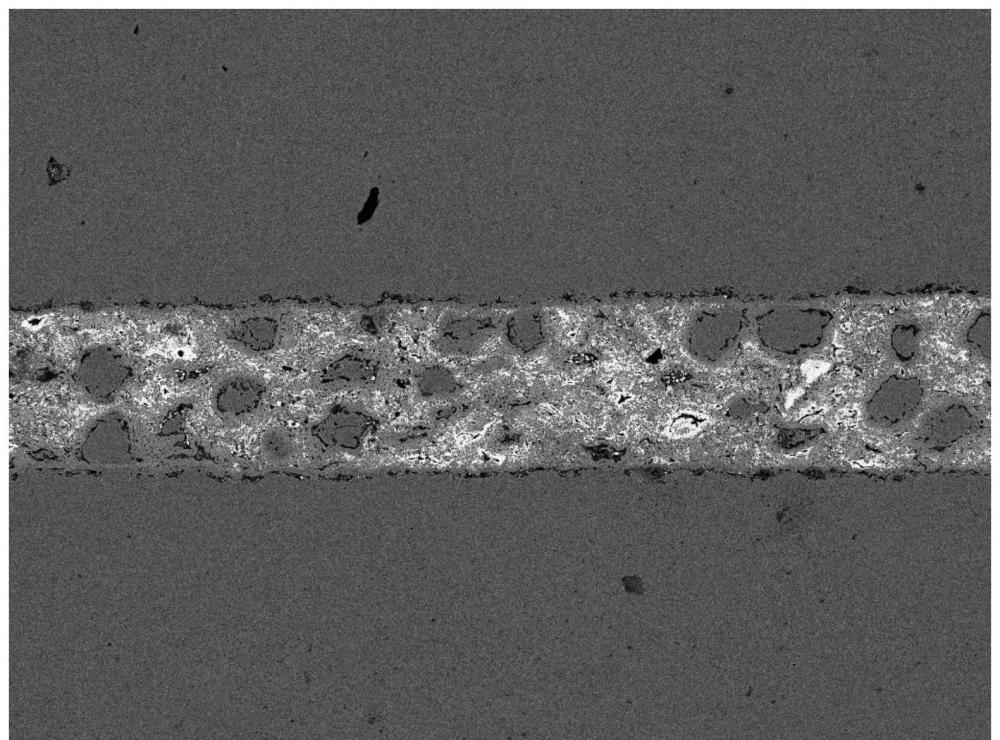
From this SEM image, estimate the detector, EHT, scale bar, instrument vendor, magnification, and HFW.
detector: BSE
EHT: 20 kV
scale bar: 100000 nm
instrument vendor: Tescan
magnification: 0.5 K X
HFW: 558 µm